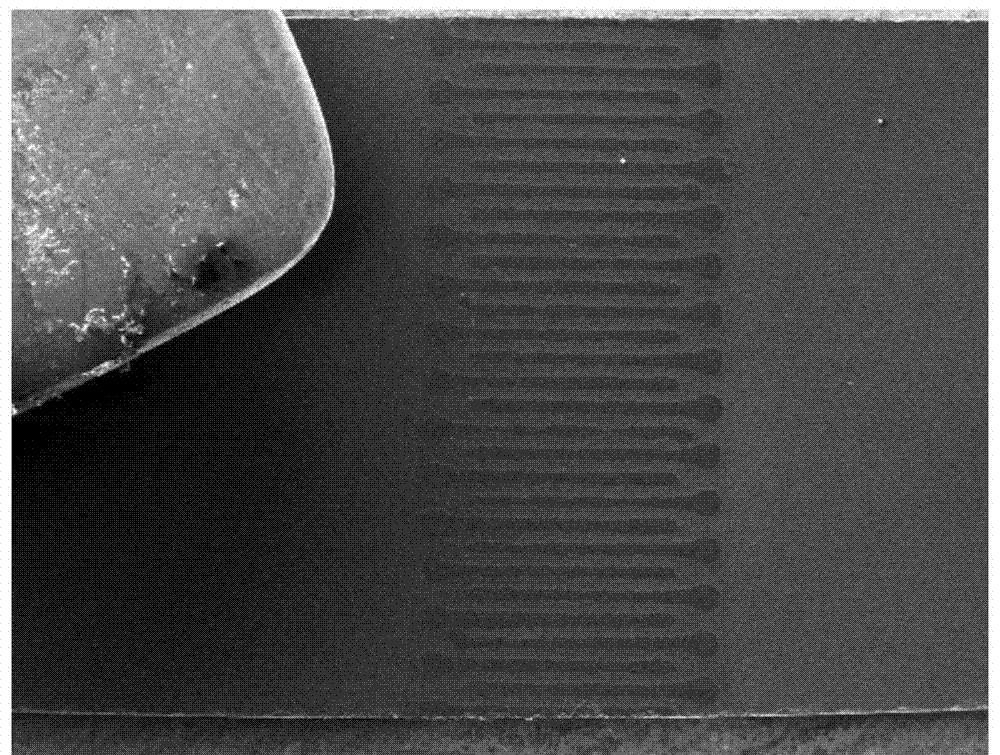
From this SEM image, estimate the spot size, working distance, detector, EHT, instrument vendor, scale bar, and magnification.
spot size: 7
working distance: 18.1 mm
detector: CDM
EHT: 30 kV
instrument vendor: FEI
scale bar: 1000000 nm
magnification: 0.025 K X